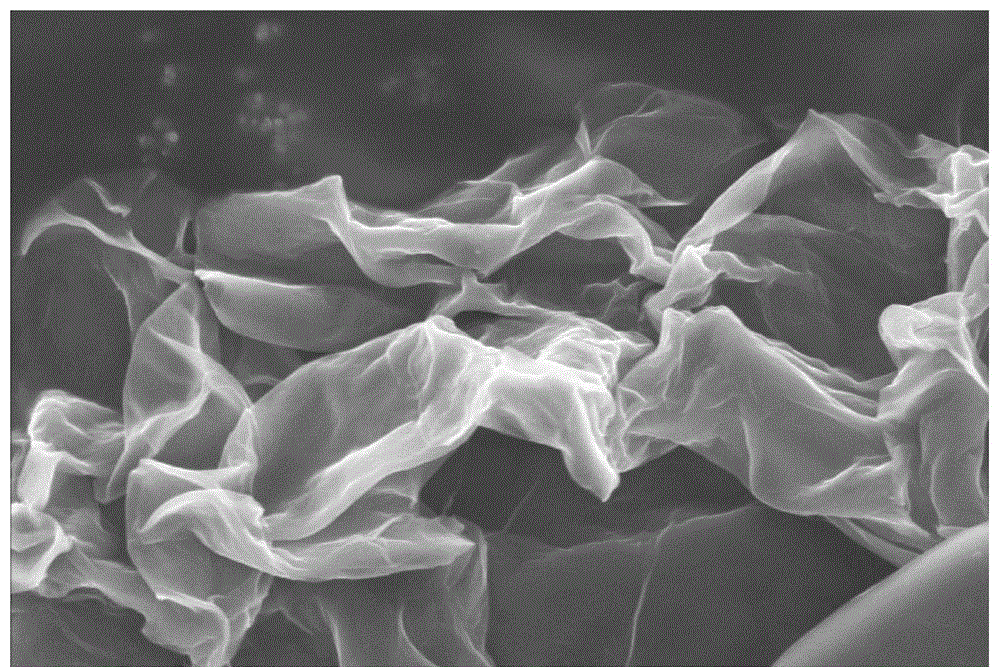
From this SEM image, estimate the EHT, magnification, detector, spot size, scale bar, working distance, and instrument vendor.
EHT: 5 kV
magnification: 50 K X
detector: TLD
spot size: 3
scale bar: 4000 nm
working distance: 6.1 mm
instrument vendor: FEI